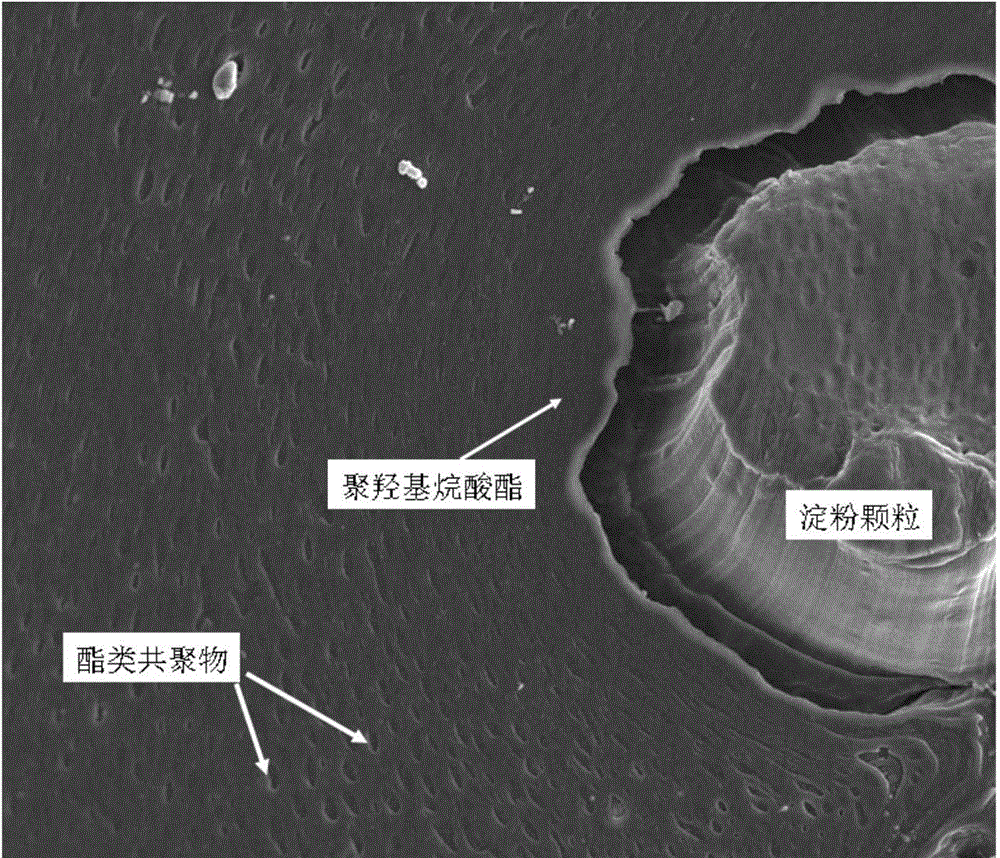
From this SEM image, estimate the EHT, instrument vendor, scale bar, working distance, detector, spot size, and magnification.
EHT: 10 kV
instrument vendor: FEI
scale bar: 50000 nm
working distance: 10.2 mm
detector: SE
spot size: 5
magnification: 2 K X